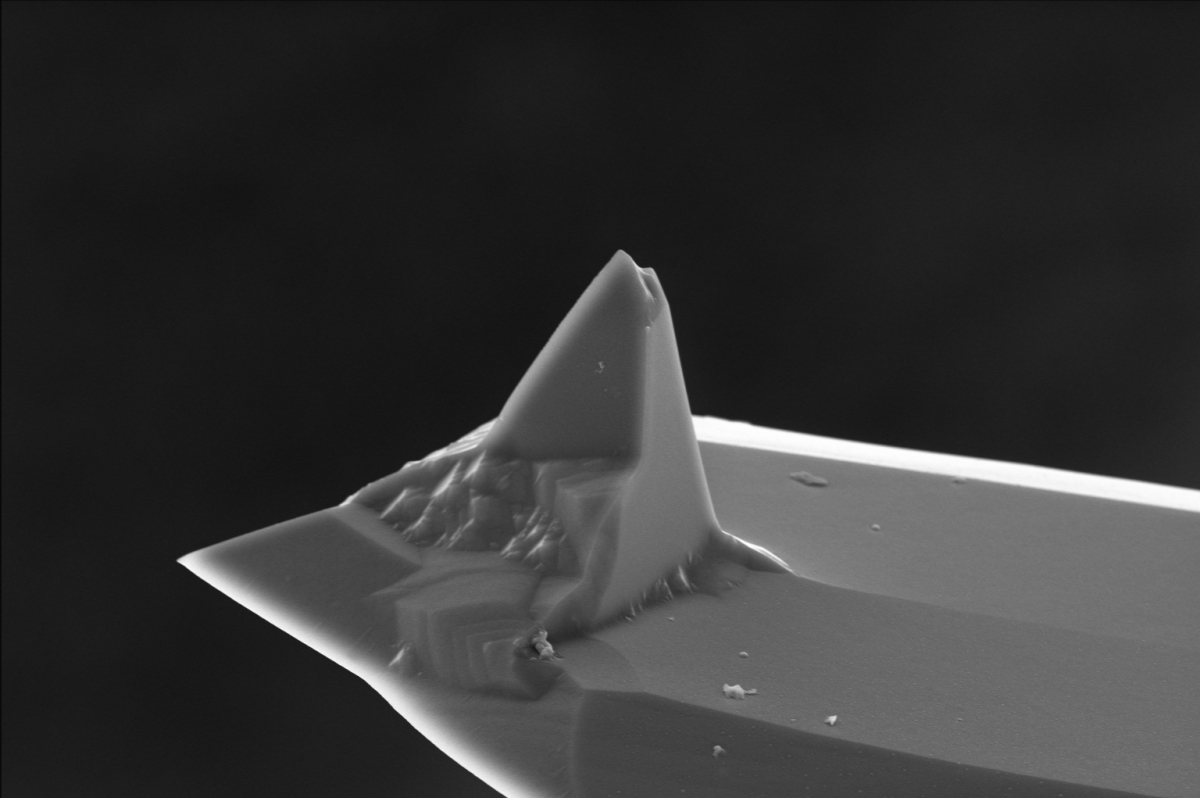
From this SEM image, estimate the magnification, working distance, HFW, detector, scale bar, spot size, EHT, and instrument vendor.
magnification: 4 K X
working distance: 10.6 mm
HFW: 51.8 µm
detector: ETD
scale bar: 10000 nm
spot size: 3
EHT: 10 kV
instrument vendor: FEI